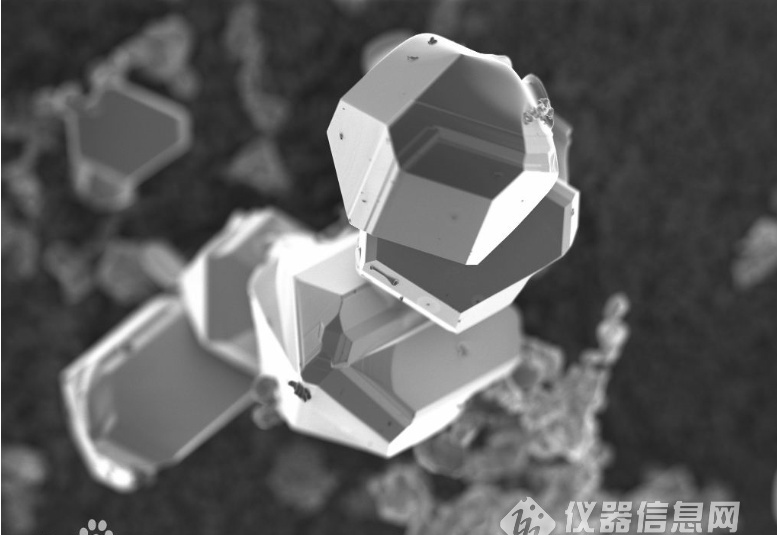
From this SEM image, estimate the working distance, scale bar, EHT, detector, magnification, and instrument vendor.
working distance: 3.9 mm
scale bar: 20000 nm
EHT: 2 kV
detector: InLens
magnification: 1 K X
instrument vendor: Zeiss